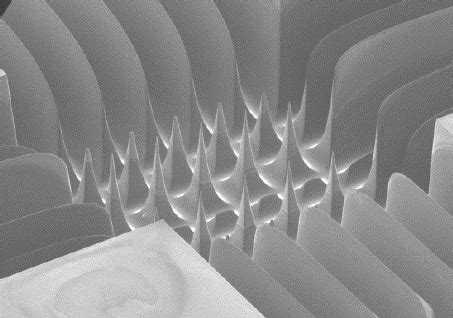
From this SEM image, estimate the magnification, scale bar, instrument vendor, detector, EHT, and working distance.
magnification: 0.035 K X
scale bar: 1000000 nm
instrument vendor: Hitachi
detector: SE(M)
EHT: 10 kV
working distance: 12 mm